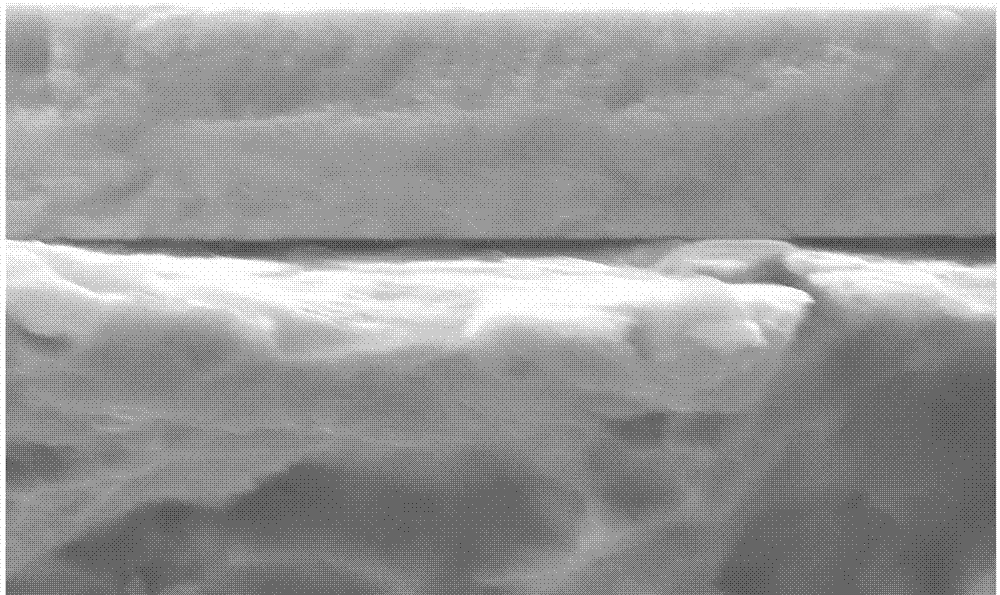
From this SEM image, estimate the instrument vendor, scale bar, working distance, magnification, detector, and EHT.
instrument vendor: Zeiss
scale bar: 1000 nm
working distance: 7.6 mm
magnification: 10 K X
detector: SE2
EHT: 15 kV